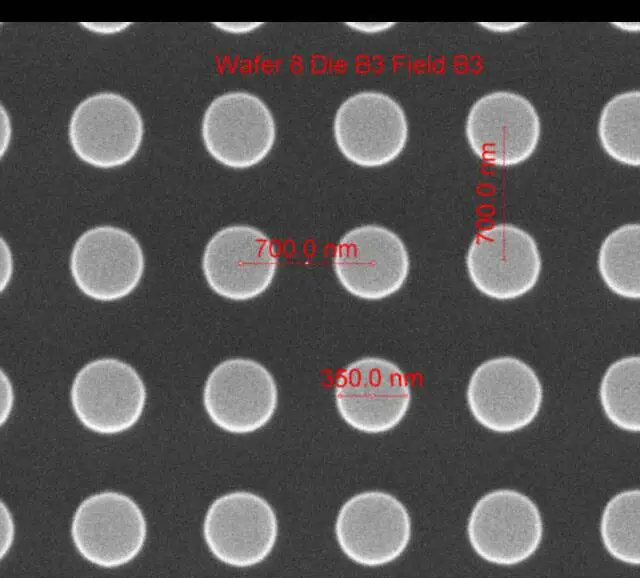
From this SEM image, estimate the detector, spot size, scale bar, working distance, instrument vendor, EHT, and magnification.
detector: ETD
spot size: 1.5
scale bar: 1000 nm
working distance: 10.3 mm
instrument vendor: FEI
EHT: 10 kV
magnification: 75 K X